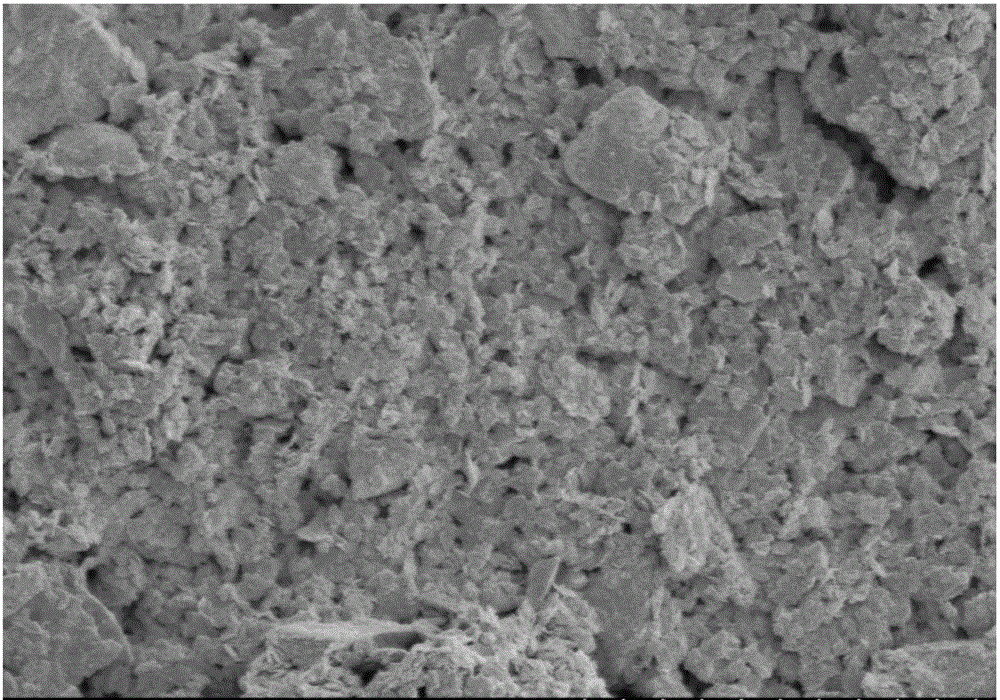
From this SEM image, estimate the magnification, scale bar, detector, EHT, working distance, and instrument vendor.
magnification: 10 K X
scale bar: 5000 nm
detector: SE(M)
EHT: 3 kV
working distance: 9.3 mm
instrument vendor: Hitachi